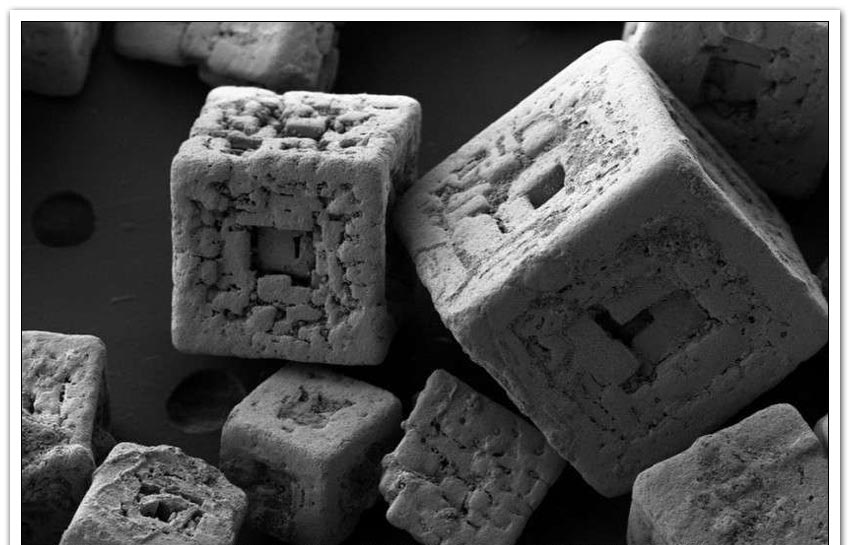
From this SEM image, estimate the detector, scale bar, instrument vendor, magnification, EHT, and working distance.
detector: SE2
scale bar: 100000 nm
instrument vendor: Zeiss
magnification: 0.15 K X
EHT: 3 kV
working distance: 6.5 mm